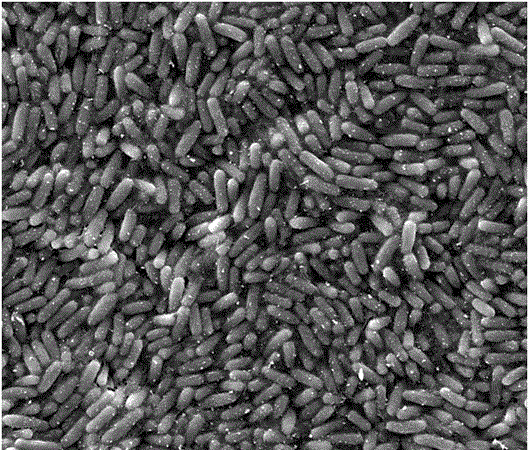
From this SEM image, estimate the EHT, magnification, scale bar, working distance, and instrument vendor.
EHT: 8 kV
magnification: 5 K X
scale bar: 10000 nm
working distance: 8.7 mm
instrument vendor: FEI